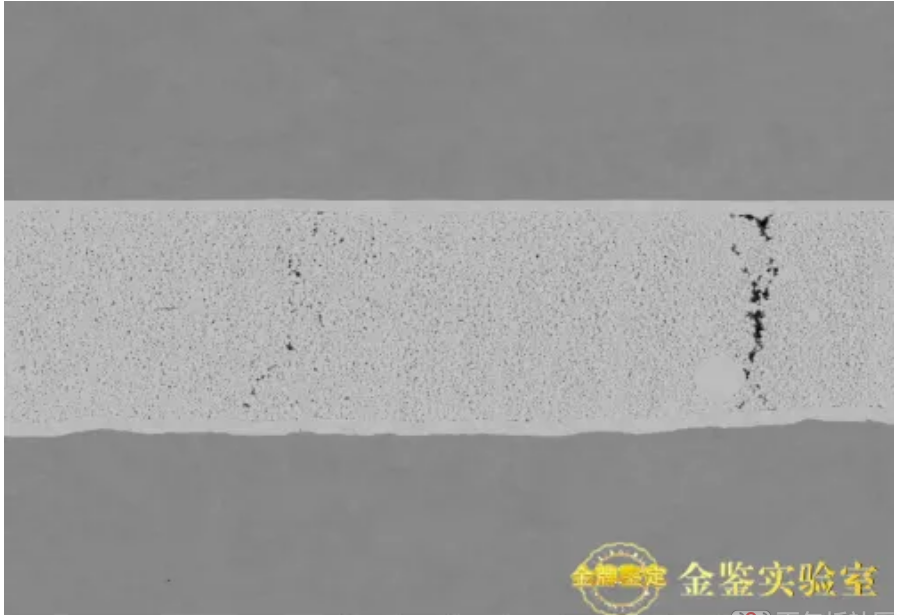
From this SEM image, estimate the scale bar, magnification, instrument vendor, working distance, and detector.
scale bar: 100000 nm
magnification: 1 K X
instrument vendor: Hitachi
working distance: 7.5 mm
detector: NL